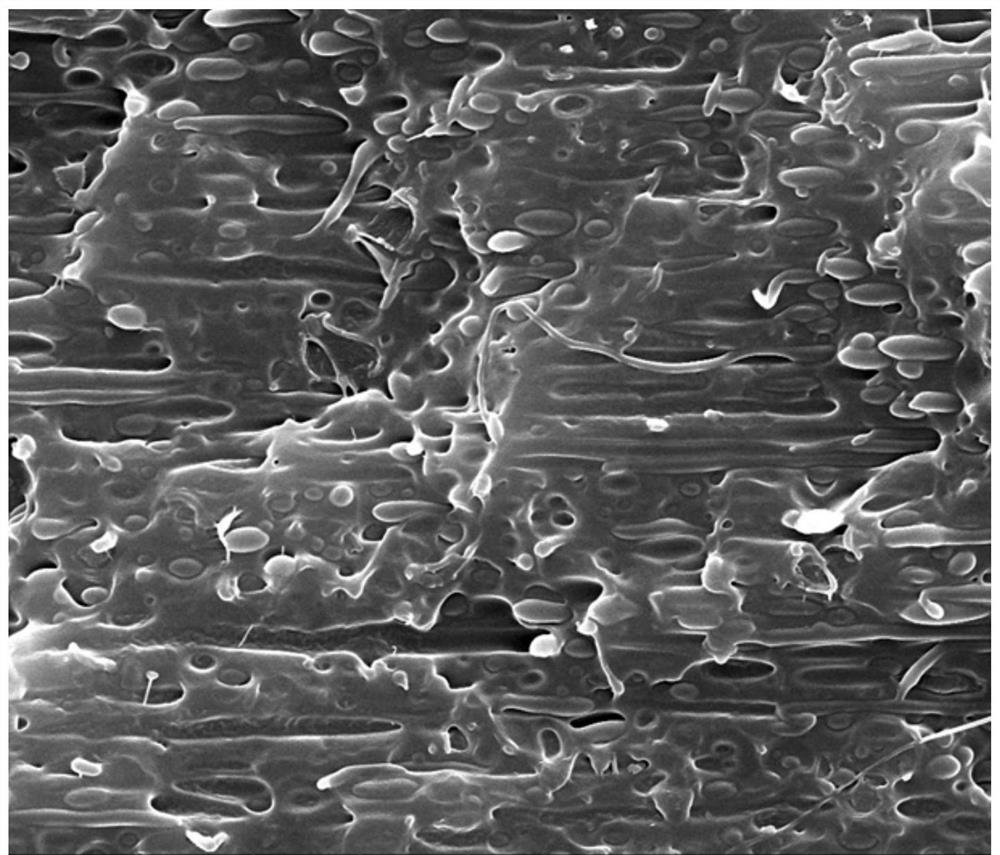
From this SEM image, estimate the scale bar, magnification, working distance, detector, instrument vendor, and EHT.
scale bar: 10000 nm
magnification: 10 K X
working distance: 12.4 mm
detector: SE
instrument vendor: FEI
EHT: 20 kV